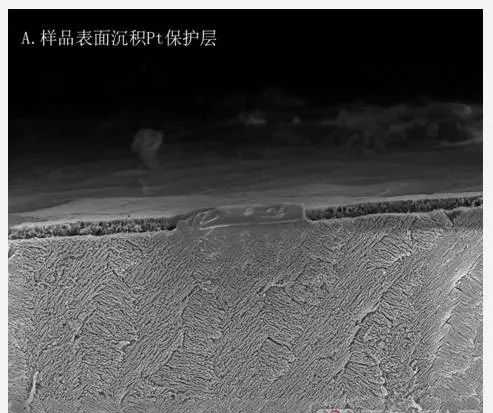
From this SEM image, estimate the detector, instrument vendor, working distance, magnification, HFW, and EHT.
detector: ETD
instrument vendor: FEI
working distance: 4 mm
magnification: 7 K X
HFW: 36.6 µm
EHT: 5 kV